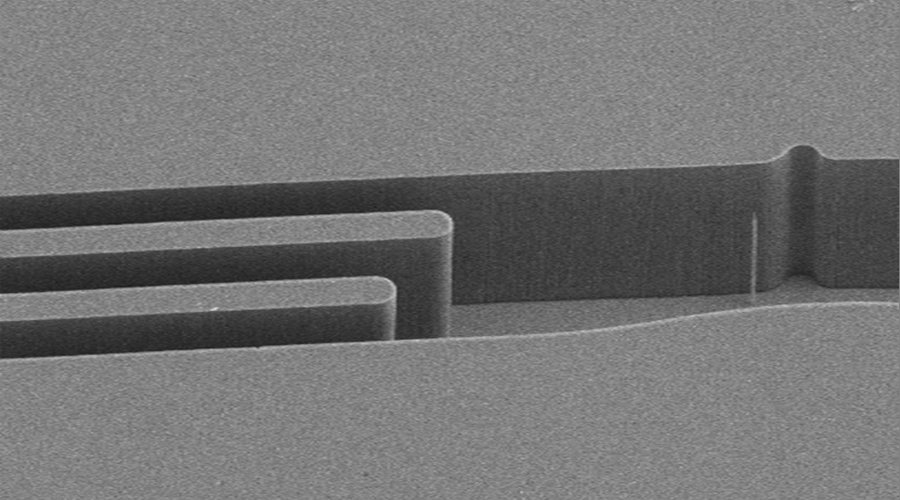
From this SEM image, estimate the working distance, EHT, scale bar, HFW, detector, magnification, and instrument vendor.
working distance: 5.84 mm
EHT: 15 kV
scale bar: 50000 nm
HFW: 283 µm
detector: SE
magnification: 1 K X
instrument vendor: other